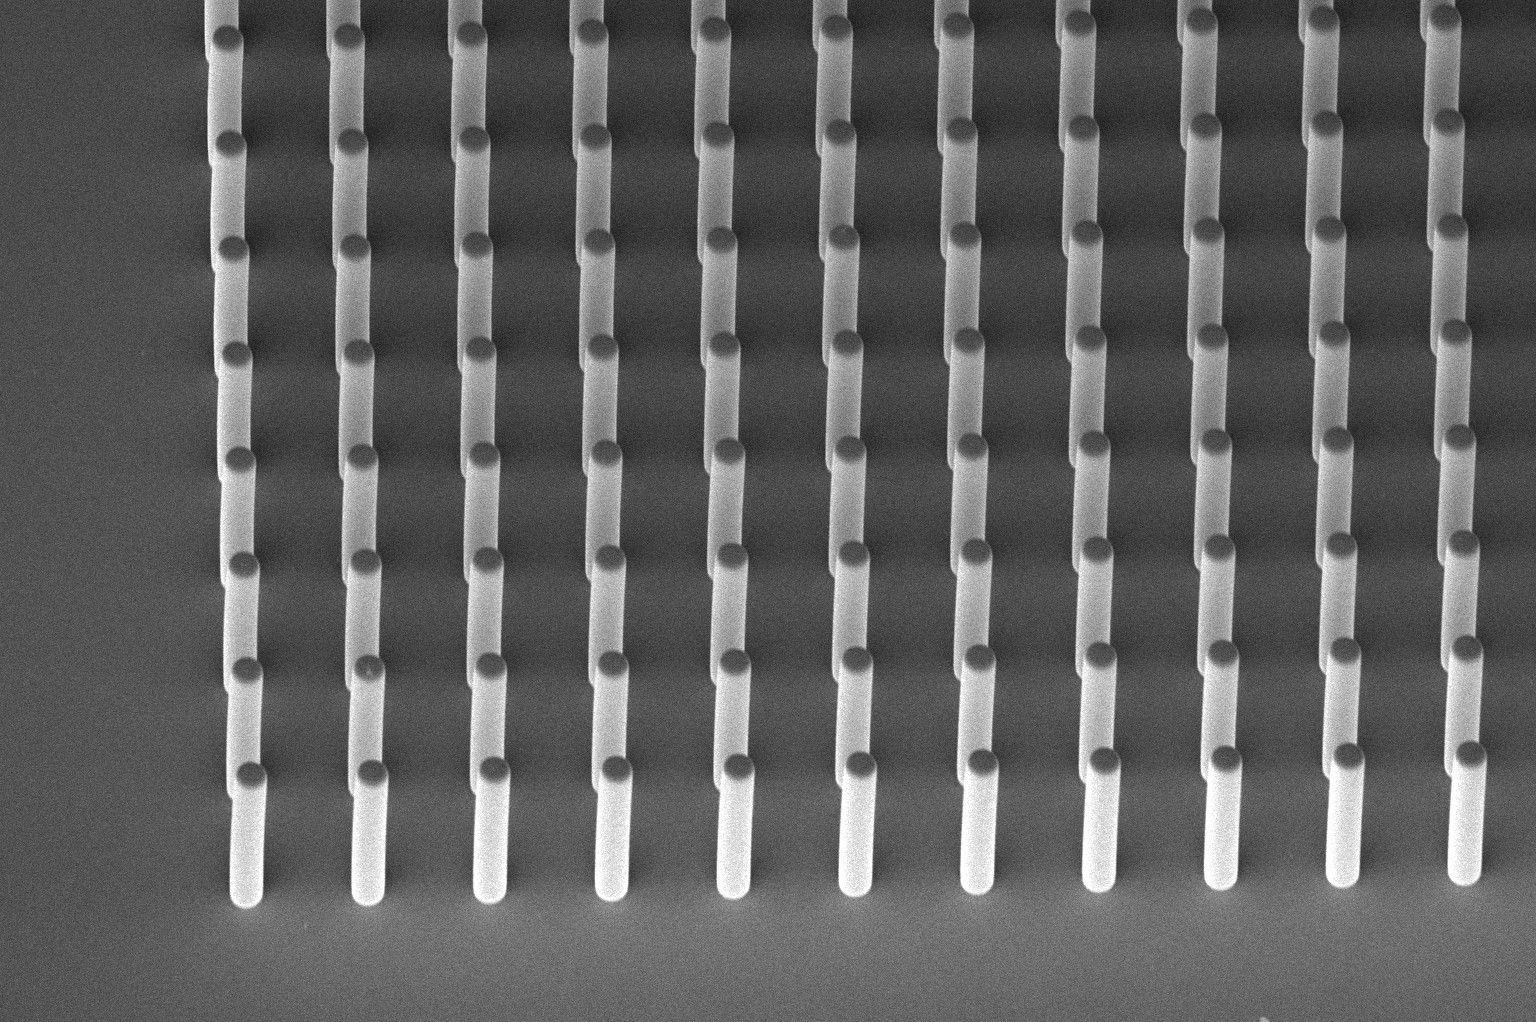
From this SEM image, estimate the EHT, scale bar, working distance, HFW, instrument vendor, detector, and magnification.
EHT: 2 kV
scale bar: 50000 nm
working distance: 3.9 mm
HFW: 318 µm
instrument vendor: FEI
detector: TLD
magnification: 0.651 K X